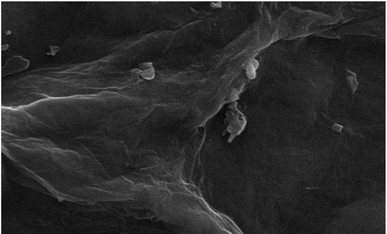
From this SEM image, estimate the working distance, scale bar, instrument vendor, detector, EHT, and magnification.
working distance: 9.5 mm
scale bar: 1000 nm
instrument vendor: Zeiss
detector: SE1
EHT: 20 kV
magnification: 10 K X